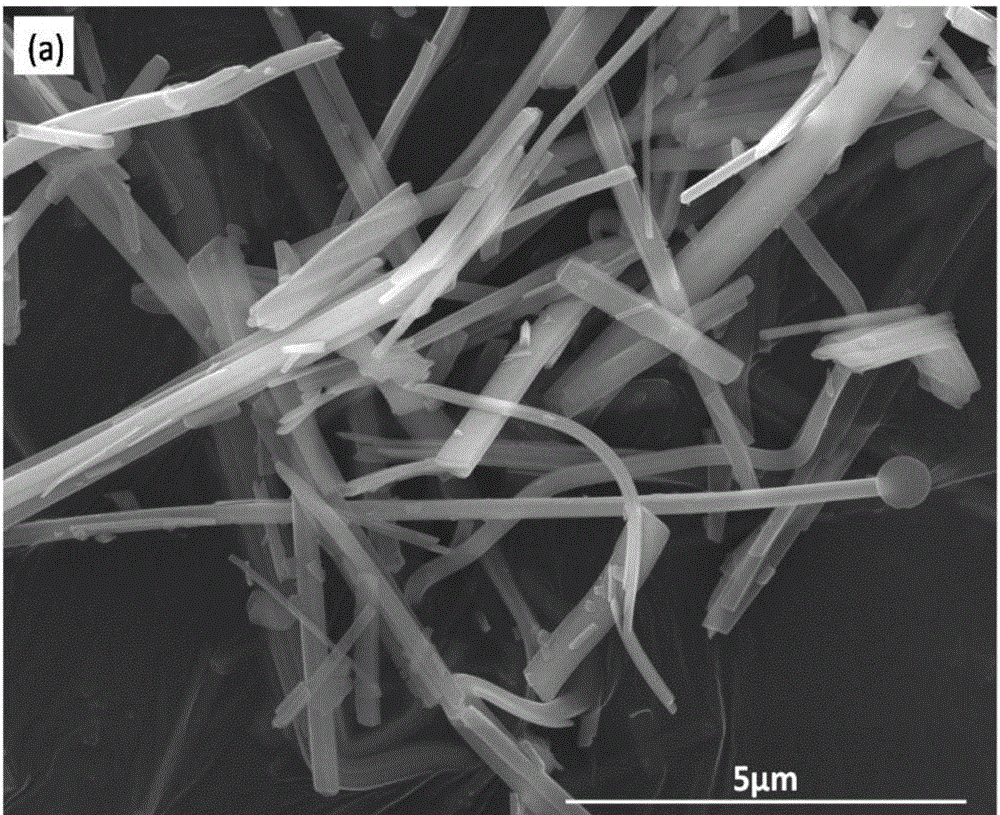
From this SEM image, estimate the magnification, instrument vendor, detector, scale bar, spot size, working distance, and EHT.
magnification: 24 K X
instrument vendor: FEI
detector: ETD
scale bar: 5000 nm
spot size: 4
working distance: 9.6 mm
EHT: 20 kV